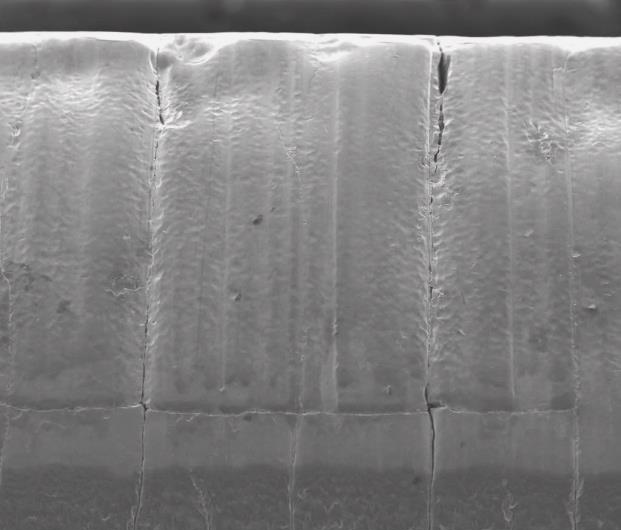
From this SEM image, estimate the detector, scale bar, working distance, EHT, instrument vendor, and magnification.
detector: ETD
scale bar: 500000 nm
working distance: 10.6 mm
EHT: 25 kV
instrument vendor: FEI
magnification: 0.15 K X